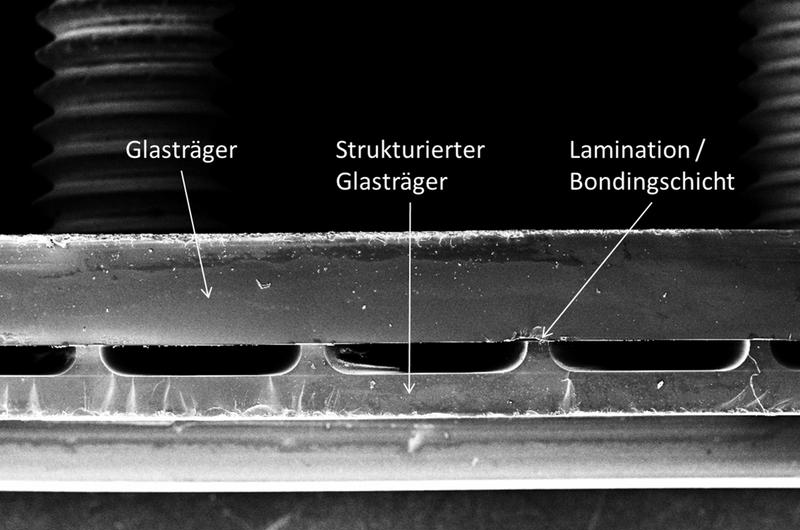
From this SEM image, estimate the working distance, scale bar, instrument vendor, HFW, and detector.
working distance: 20.2 mm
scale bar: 2000000 nm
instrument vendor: other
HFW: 11560 µm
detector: SE